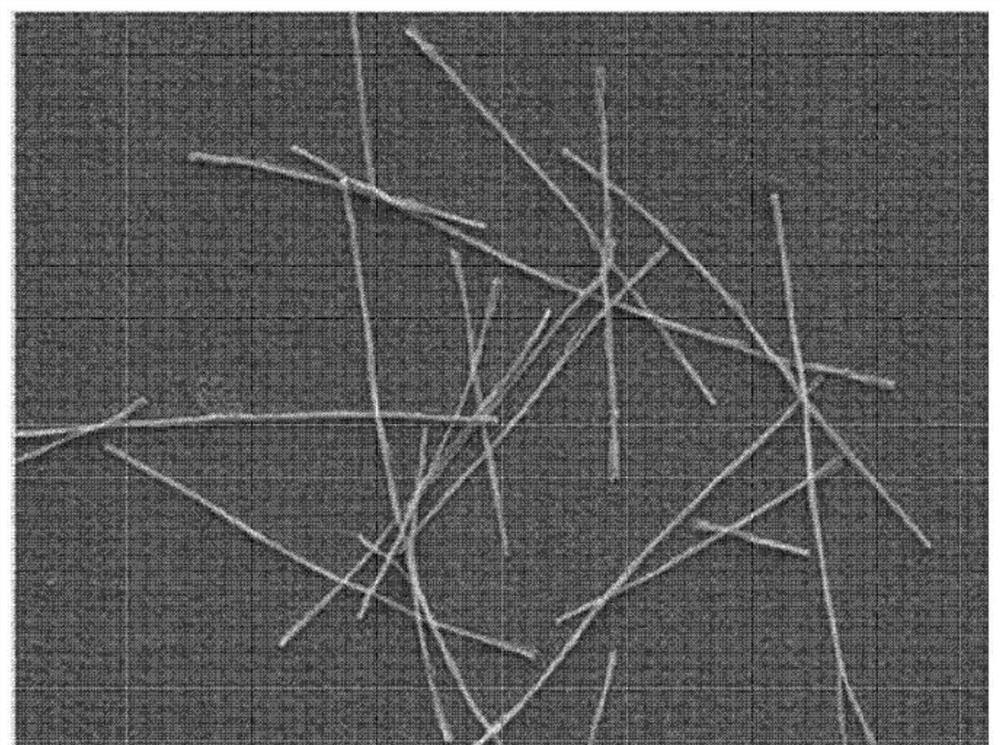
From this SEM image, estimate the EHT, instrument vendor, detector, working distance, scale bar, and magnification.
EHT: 5 kV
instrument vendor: JEOL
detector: LED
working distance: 10 mm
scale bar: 10000 nm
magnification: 2 K X